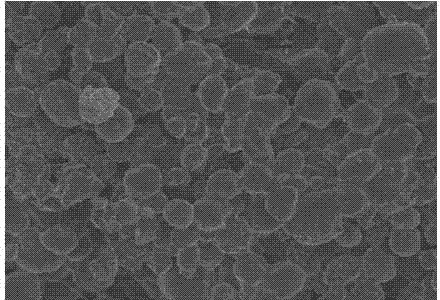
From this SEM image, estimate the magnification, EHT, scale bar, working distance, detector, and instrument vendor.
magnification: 0.4 K X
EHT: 5 kV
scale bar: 100000 nm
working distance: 8 mm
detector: SE(M)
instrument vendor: Hitachi